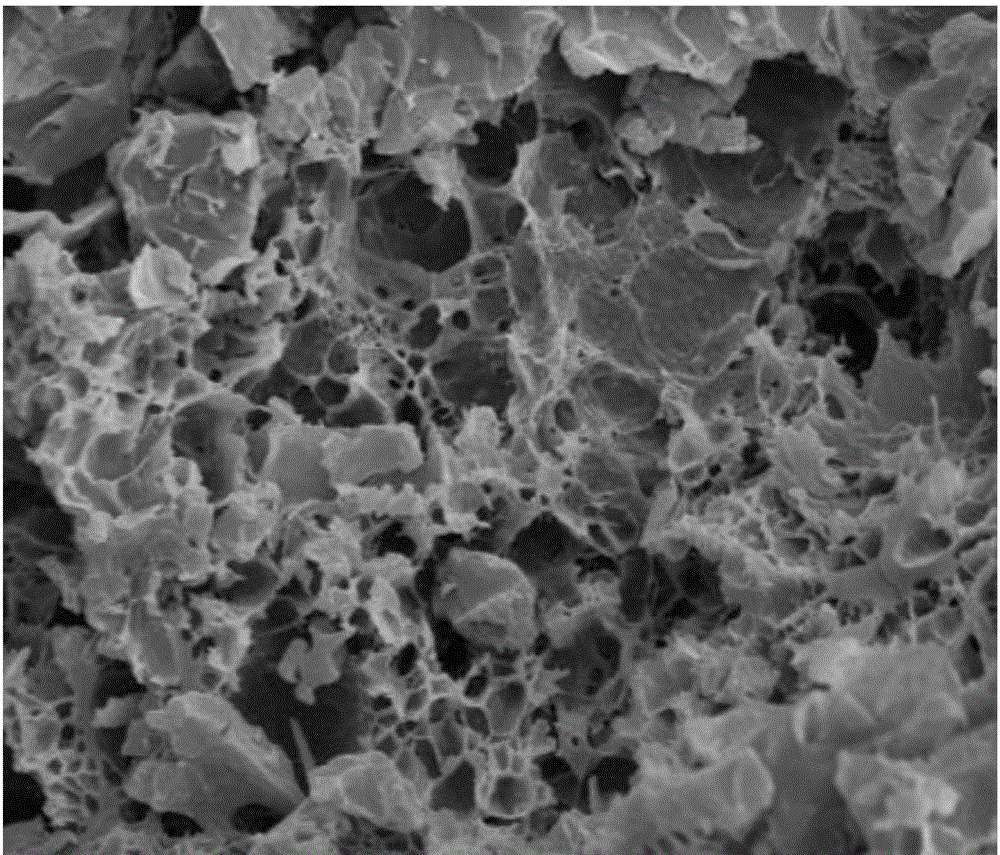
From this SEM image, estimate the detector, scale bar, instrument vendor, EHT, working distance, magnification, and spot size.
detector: ETD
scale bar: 10000 nm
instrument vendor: FEI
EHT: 12.5 kV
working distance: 9.6 mm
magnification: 3 K X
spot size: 4.5